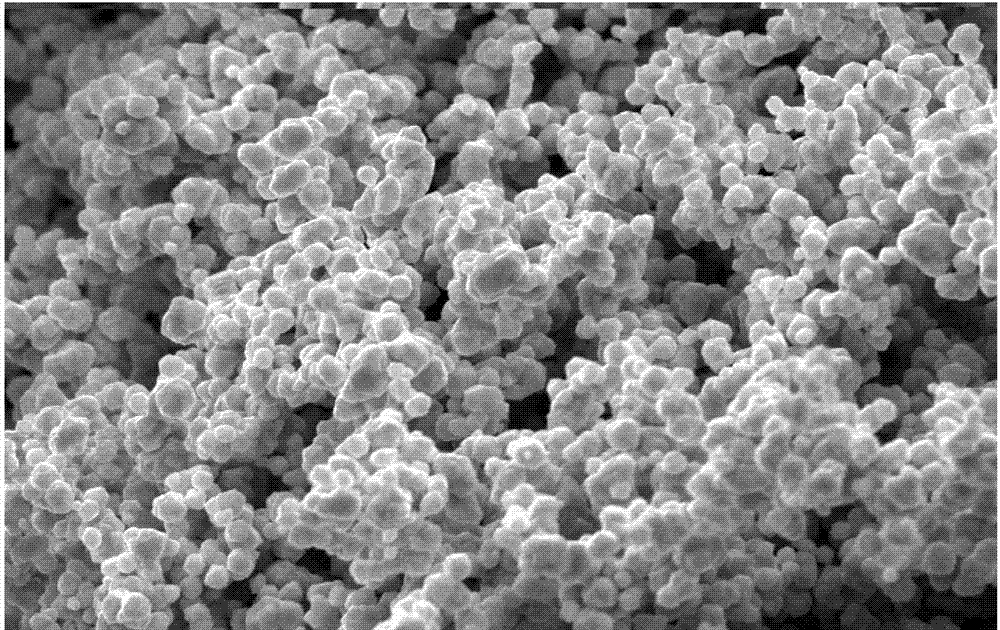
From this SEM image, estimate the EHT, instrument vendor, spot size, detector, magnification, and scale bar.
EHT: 20 kV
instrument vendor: FEI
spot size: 3.6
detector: SE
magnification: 3 K X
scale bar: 10000 nm